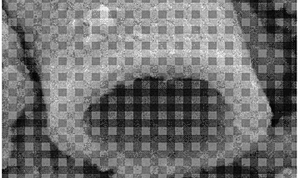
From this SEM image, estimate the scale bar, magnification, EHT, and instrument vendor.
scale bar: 1000 nm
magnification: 13 K X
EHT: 15 kV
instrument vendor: JEOL